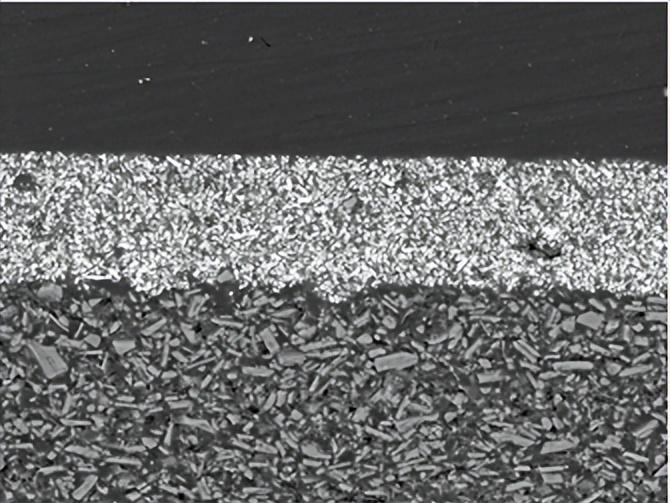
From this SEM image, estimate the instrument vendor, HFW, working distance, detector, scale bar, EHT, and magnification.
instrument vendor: Tescan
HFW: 185 µm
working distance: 14.28 mm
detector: BSE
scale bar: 50000 nm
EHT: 20 kV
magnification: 1.49 K X